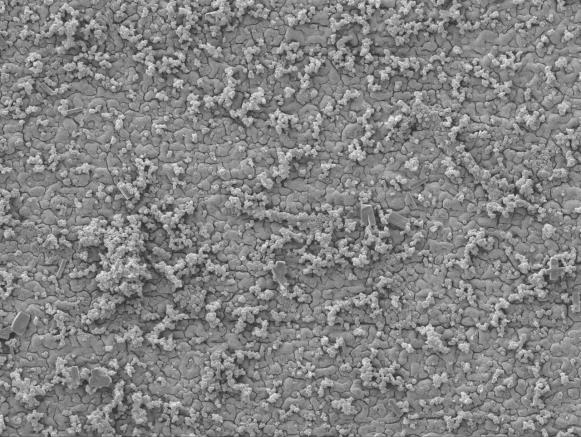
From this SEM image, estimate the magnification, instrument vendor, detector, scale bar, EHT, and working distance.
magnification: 0.8 K X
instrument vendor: JEOL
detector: SEI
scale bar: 10000 nm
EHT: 10 kV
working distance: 8.4 mm